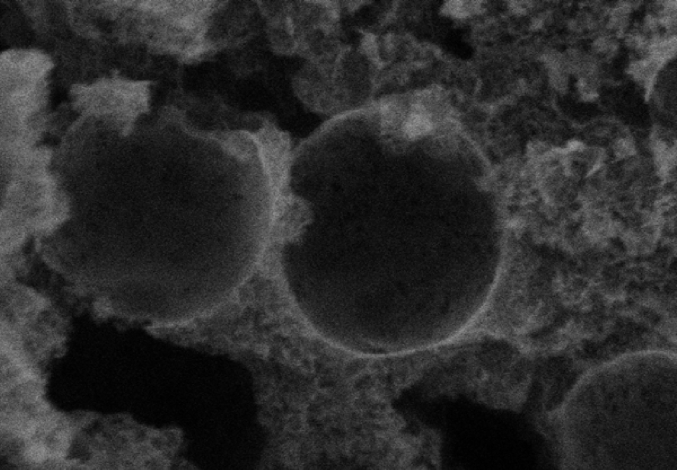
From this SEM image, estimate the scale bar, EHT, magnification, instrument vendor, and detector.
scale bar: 500 nm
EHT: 2 kV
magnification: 100 K X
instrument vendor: Hitachi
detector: SE